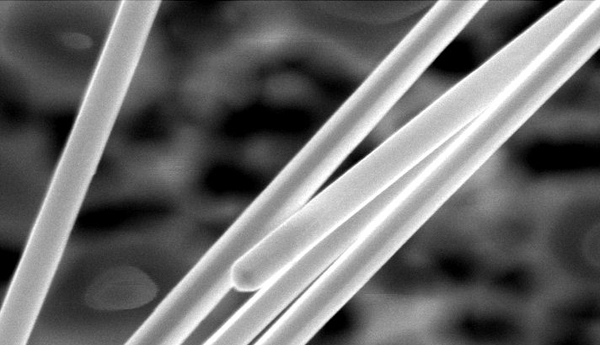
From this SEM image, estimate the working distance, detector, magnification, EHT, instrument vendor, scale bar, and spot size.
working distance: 6 mm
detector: TLD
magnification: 40 K X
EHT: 10 kV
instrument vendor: FEI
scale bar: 500 nm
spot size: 3